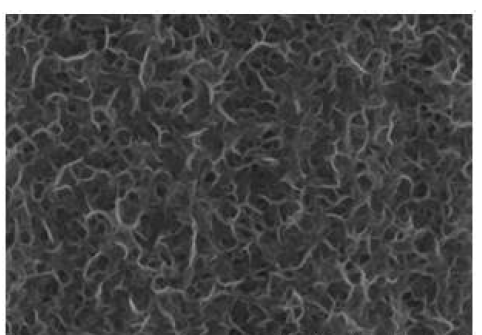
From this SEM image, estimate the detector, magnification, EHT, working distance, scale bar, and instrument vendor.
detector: SE(UL)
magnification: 100 K X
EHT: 3 kV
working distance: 9.1 mm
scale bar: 500 nm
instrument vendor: Hitachi